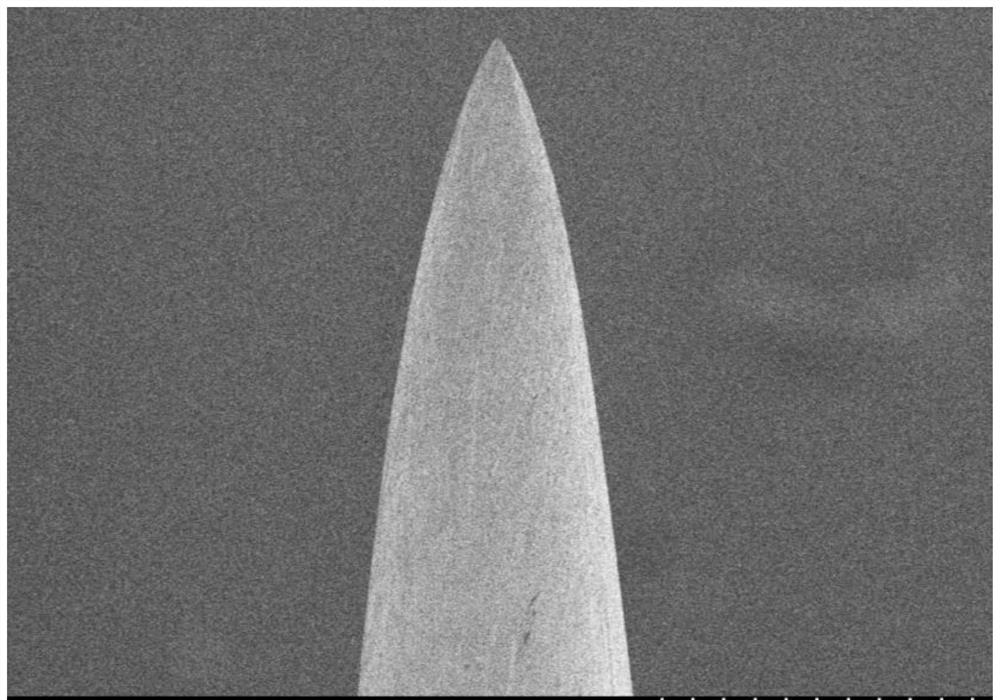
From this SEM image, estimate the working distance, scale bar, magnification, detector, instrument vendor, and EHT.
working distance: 8.2 mm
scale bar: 100000 nm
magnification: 0.4 K X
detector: SE(M)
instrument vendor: Hitachi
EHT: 1 kV